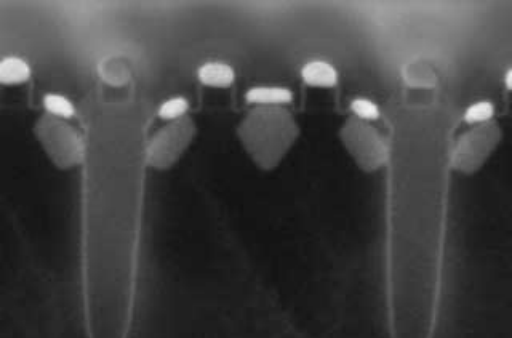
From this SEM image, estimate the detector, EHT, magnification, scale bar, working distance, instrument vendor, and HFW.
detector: MD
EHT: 2 kV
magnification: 200 K X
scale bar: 300 nm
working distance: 1.5 mm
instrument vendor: FEI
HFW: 0.635 µm